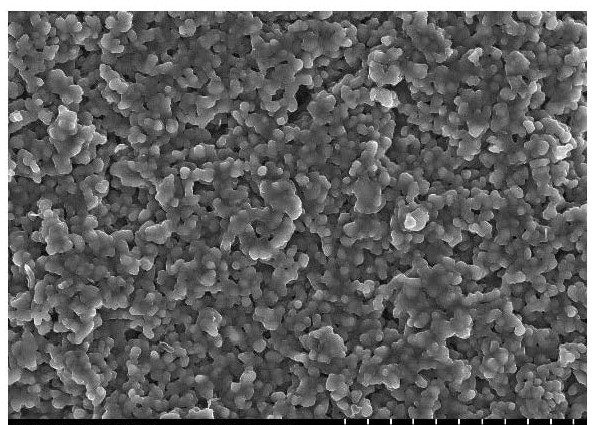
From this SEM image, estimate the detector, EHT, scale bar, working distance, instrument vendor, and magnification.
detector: SE(UL)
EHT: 10 kV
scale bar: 10000 nm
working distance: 8.9 mm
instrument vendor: Hitachi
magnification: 5 K X